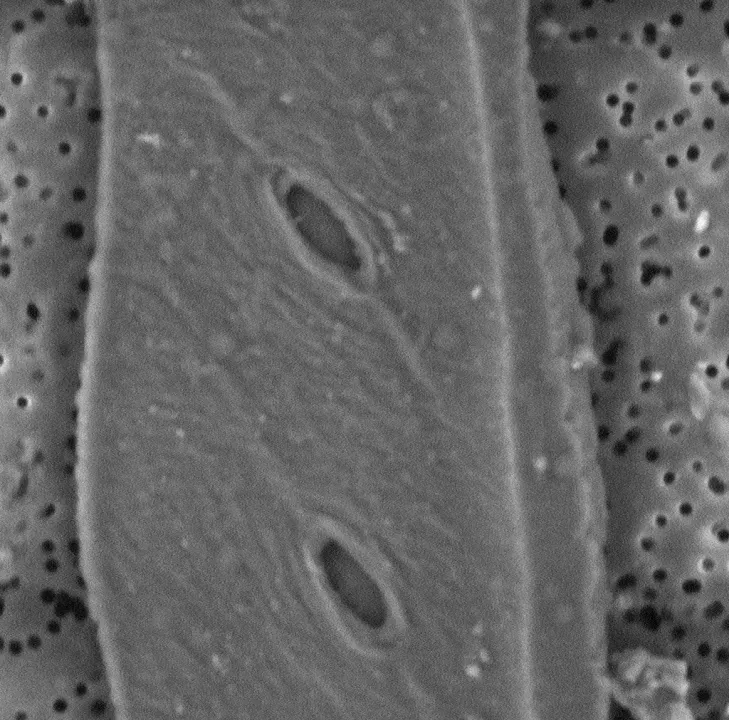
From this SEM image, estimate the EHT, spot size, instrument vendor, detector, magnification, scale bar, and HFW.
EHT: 5 kV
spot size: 3.7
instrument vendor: Thermo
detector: BSD Full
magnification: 13.5 K X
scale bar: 5000 nm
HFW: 19.9 µm